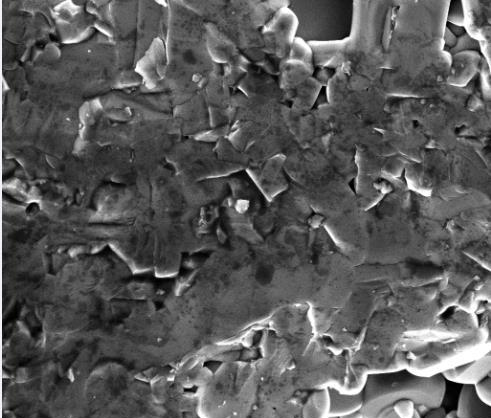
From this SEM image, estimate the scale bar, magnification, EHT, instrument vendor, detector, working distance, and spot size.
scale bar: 20000 nm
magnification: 4 K X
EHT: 15 kV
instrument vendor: FEI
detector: ETD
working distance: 14.4 mm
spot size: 2